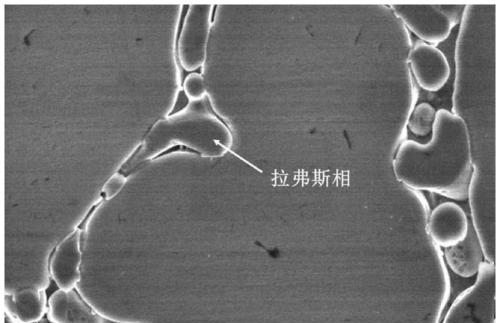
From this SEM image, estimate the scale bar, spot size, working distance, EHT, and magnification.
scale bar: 10000 nm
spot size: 3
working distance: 8.6 mm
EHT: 20 kV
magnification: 4 K X